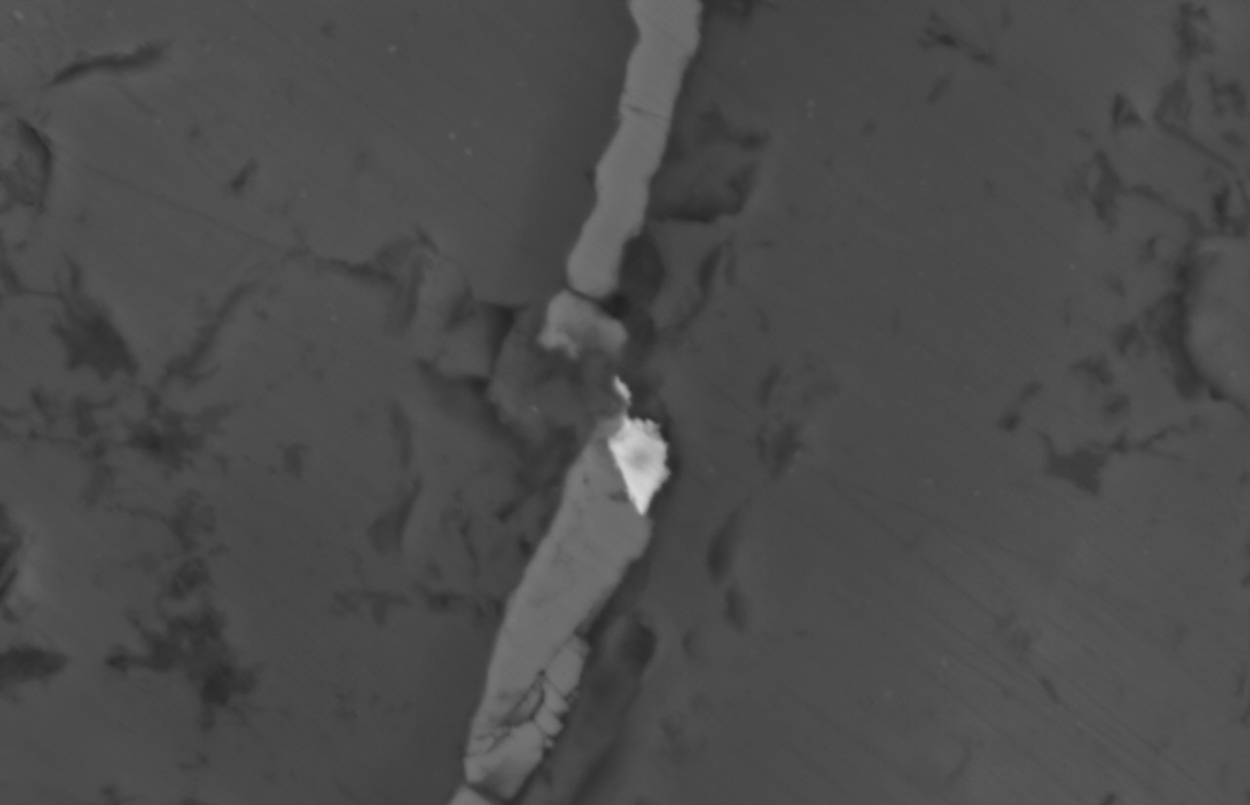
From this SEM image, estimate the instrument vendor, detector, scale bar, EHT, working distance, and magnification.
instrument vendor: Hitachi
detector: BSE3D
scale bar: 30000 nm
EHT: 20 kV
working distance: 12.8 mm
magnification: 1.9 K X